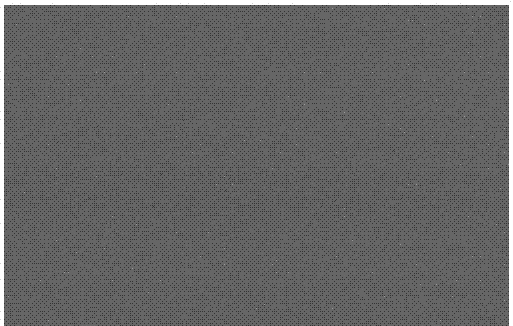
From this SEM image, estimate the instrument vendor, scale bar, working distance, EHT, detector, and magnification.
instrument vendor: Hitachi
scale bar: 500 nm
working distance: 8.2 mm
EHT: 3 kV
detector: SE(M,LA3)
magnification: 60 K X